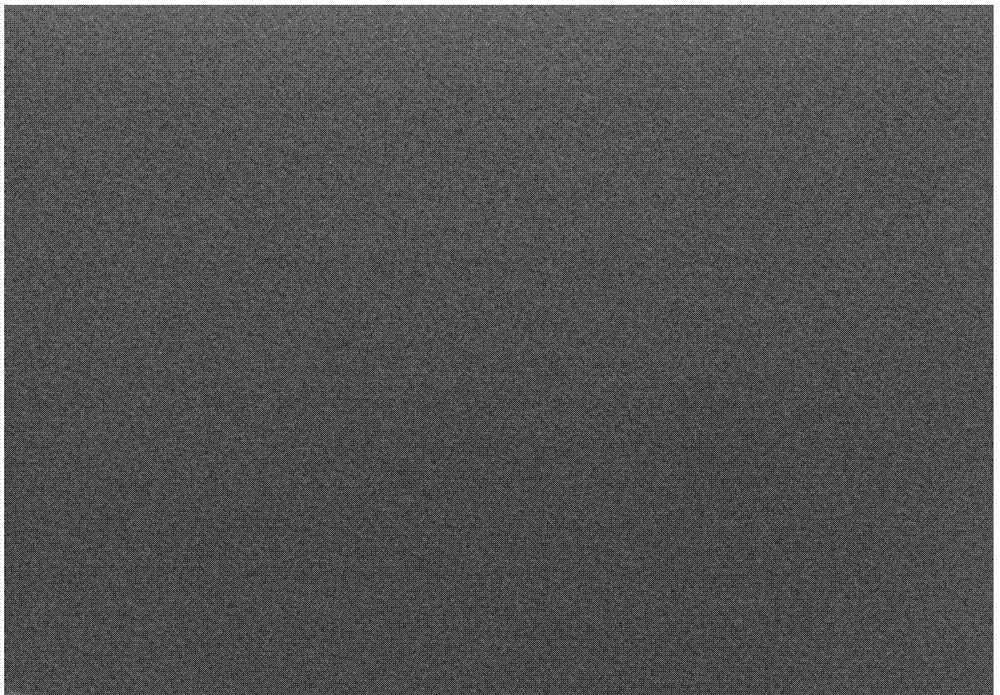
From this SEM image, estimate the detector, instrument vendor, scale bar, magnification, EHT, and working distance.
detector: SE(U)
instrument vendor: Hitachi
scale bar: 500 nm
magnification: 60 K X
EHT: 5 kV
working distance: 8.8 mm